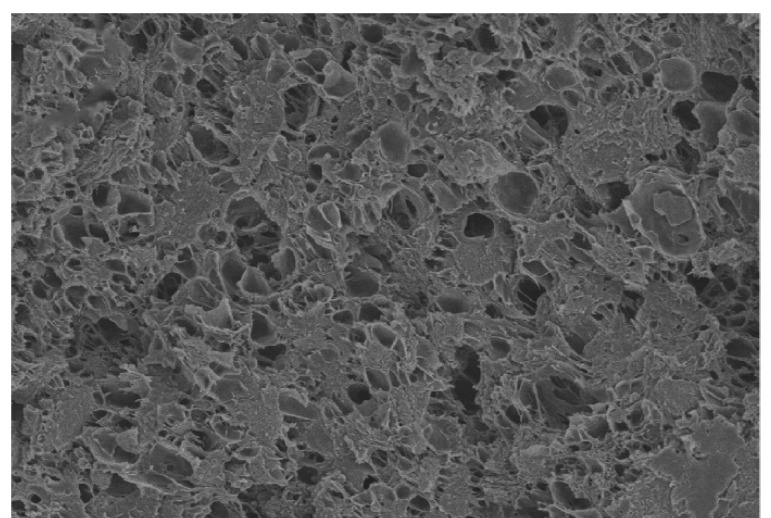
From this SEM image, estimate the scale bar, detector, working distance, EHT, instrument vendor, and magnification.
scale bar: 50000 nm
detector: SE(M)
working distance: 14.6 mm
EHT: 5 kV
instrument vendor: Hitachi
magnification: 1 K X